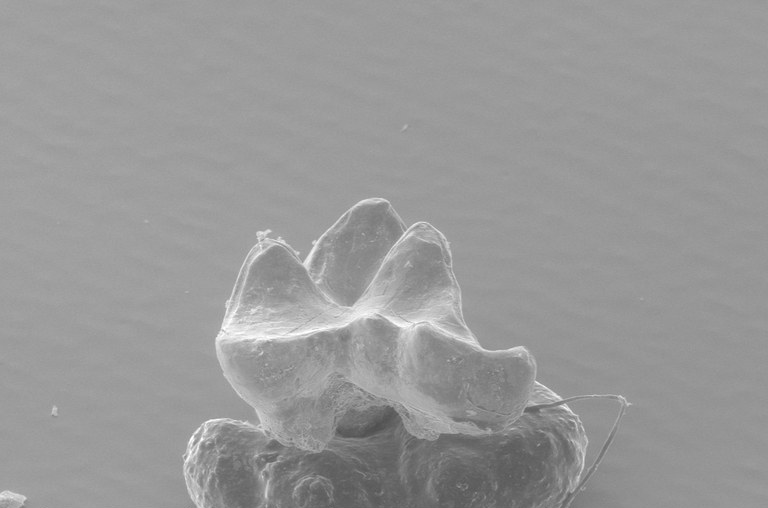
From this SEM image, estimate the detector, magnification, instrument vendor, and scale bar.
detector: AUX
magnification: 0.09 K X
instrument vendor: FEI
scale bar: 500000 nm